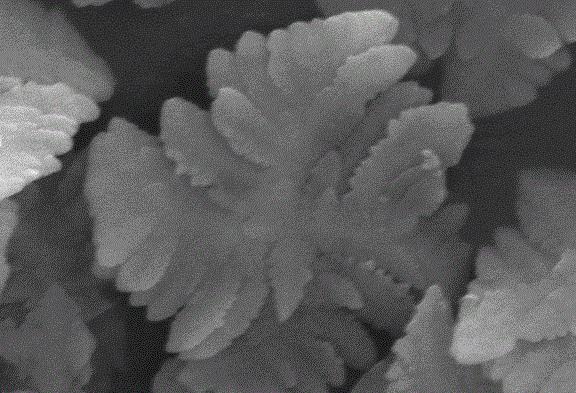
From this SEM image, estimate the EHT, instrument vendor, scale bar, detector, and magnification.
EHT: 25 kV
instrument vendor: JEOL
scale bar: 1000 nm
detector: SEI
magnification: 13 K X